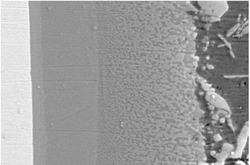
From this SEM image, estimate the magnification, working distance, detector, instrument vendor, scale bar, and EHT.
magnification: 1.1 K X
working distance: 8.4 mm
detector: SE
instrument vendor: Hitachi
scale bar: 50000 nm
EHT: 15 kV